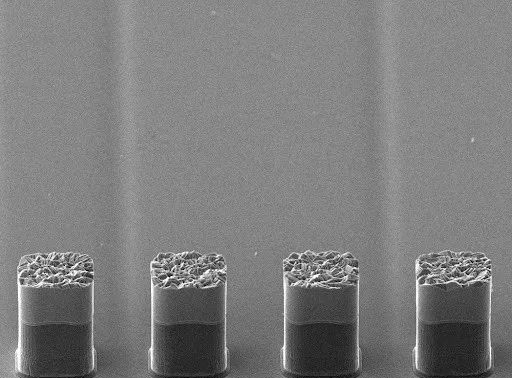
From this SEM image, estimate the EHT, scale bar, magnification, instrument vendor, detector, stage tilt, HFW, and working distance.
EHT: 20 kV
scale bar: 50000 nm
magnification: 1 K X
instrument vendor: other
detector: SE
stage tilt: -30°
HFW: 279 µm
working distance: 18.99 mm